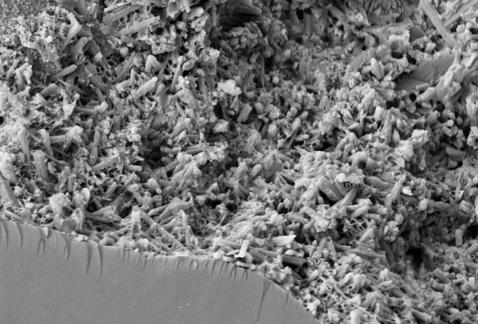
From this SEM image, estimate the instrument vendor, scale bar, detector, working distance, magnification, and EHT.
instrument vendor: Zeiss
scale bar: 1000 nm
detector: MPSE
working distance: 8 mm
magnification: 10 K X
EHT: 4 kV